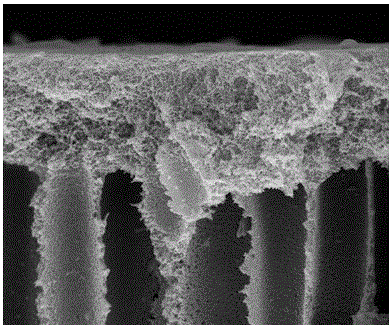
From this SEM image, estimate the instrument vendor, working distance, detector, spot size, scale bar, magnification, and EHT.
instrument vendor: FEI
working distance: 9.2 mm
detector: ETD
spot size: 3.5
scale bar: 5000 nm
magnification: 5 K X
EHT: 15 kV